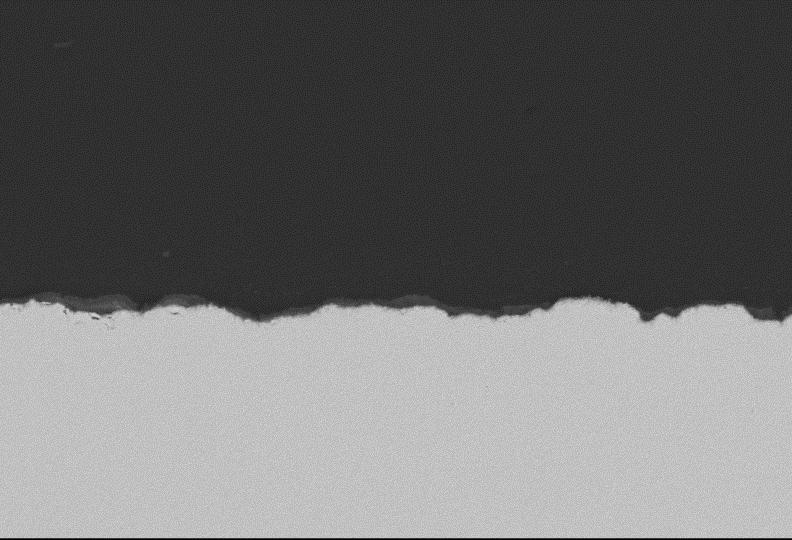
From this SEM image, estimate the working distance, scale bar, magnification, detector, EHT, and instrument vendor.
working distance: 10 mm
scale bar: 50000 nm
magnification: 0.5 K X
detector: BEC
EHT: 20 kV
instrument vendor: JEOL